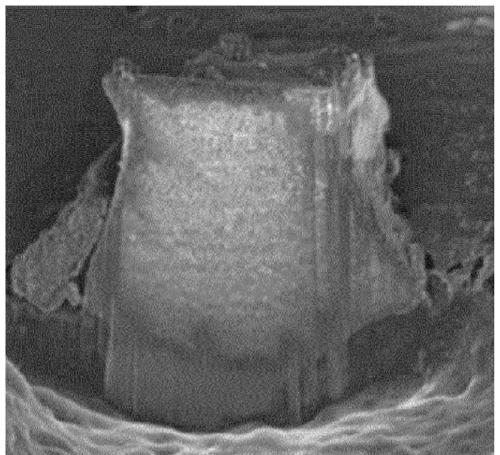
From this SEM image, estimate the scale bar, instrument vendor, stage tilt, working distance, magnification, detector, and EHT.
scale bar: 3000 nm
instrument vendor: FEI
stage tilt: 52°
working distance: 5 mm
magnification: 30 K X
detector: TLD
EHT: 2 kV